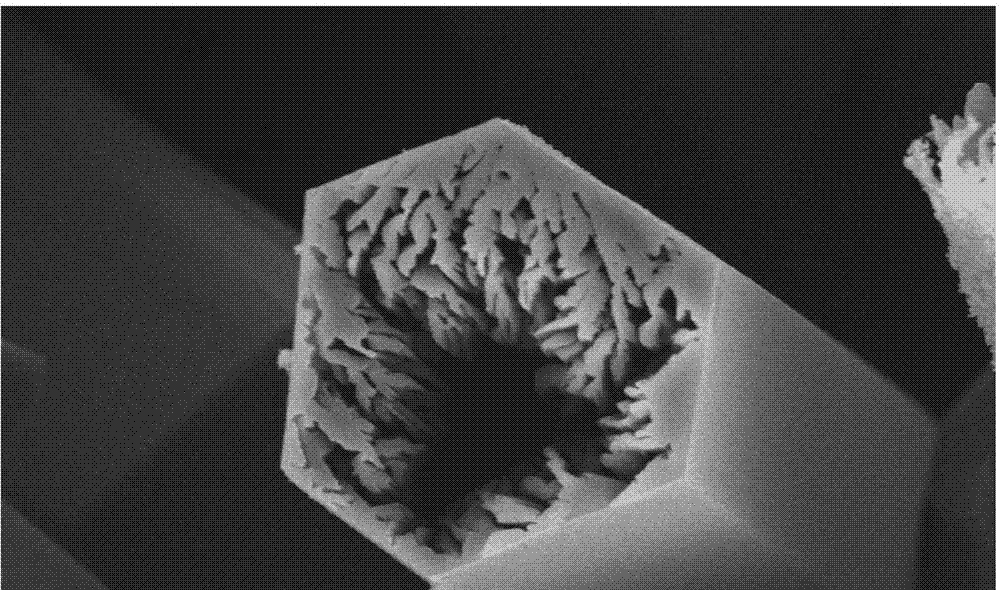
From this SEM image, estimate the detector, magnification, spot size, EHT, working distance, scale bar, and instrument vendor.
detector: SE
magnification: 3 K X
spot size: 3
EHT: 15 kV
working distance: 10.2 mm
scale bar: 10000 nm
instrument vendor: FEI